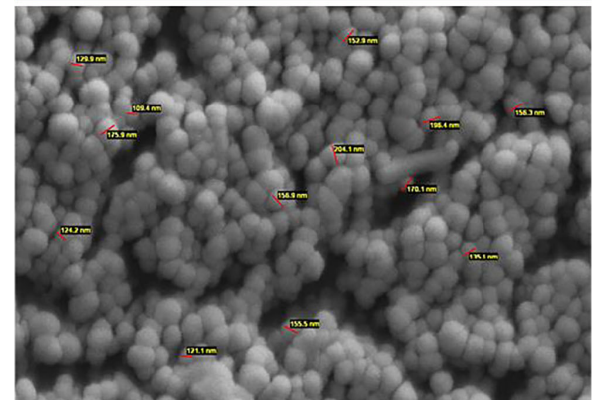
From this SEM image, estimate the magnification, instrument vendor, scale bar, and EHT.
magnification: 20 K X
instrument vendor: other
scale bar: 1000 nm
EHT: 26 kV